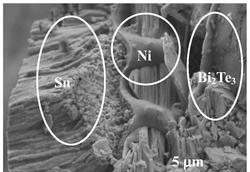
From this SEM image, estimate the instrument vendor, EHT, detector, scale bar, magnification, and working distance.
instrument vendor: Hitachi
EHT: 5 kV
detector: SE(L)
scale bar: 5000 nm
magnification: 10 K X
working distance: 13.4 mm